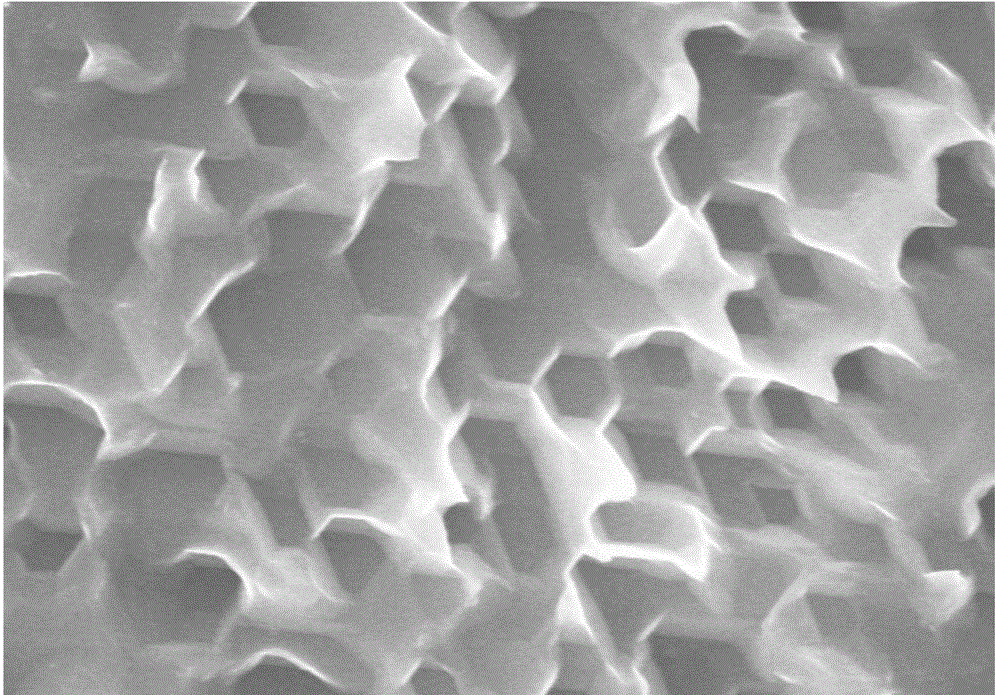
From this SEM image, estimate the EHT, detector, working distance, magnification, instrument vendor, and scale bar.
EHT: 10 kV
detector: SE(M)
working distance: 5.2 mm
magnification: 30 K X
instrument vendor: Hitachi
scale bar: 1000 nm